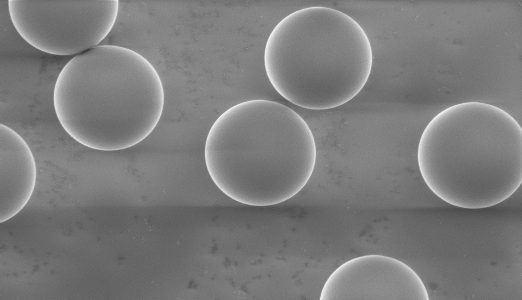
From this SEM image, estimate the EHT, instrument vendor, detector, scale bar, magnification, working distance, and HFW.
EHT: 1 kV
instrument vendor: Thermo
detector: SED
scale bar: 15000 nm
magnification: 10 K X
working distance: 7.41 mm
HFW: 51.9 µm